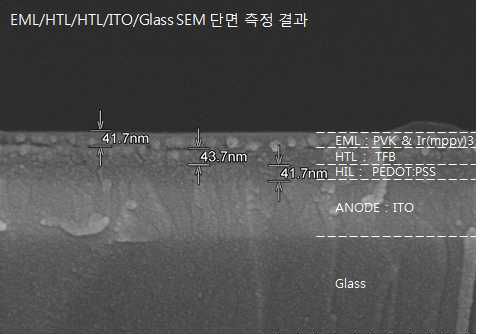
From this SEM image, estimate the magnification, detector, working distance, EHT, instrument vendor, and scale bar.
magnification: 100 K X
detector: SE(U,LA3)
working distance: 11.3 mm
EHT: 15 kV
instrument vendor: Hitachi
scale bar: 500 nm